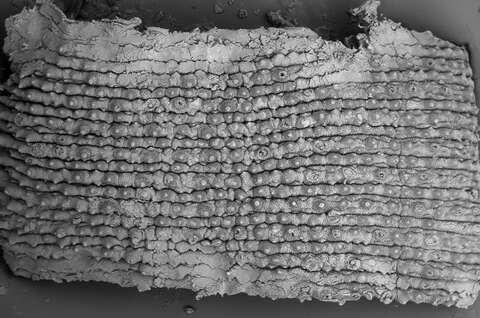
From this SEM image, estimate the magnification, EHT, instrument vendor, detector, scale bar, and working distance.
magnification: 0.081 K X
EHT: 15 kV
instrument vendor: Zeiss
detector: CZ BSD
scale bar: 200000 nm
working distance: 8 mm